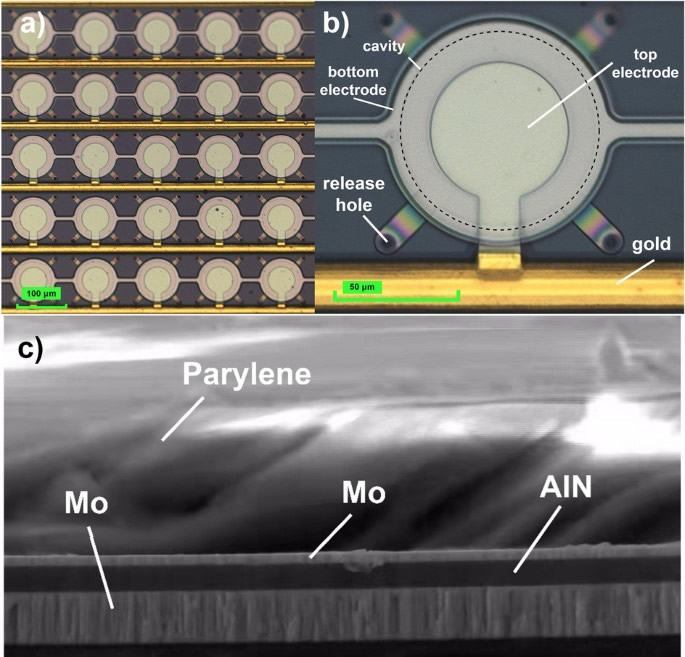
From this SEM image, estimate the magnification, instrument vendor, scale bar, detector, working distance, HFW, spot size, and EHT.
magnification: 26.665 K X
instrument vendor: FEI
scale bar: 4000 nm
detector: ETD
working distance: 10.9 mm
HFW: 11.2 µm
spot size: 3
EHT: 10 kV